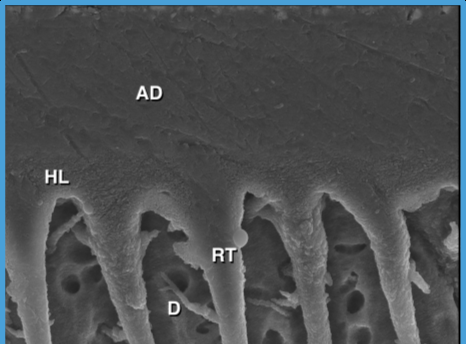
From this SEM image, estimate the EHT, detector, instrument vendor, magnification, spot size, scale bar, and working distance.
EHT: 10 kV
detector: ETD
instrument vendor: FEI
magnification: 10 K X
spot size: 2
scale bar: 10000 nm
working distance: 9.8 mm